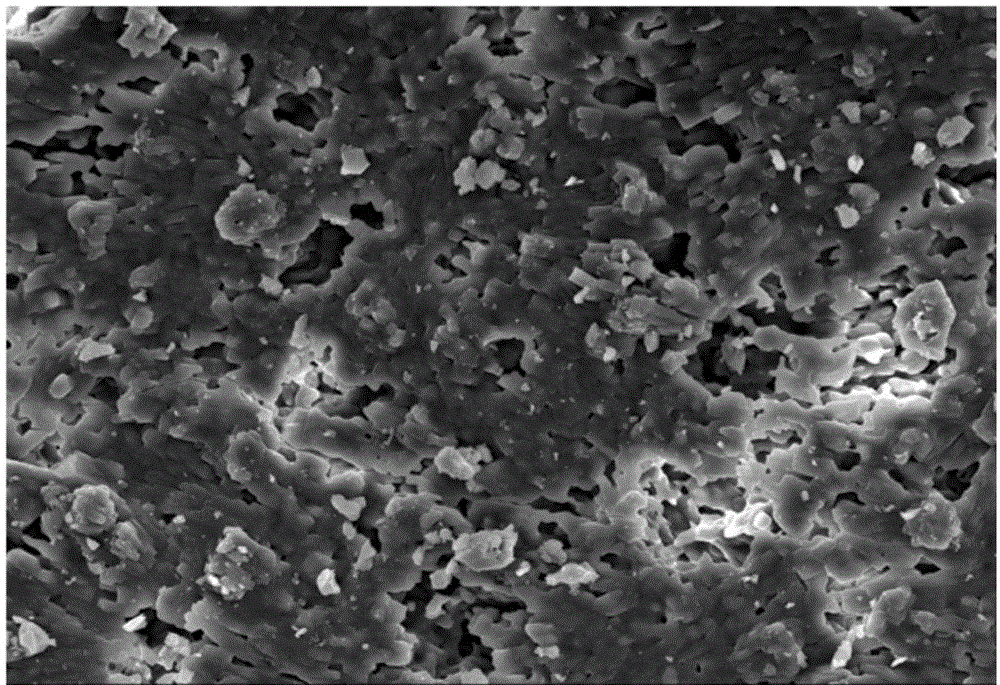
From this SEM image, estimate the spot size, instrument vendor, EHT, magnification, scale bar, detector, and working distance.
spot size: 35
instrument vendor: JEOL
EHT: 15 kV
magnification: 1 K X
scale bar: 10000 nm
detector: SEI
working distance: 13 mm